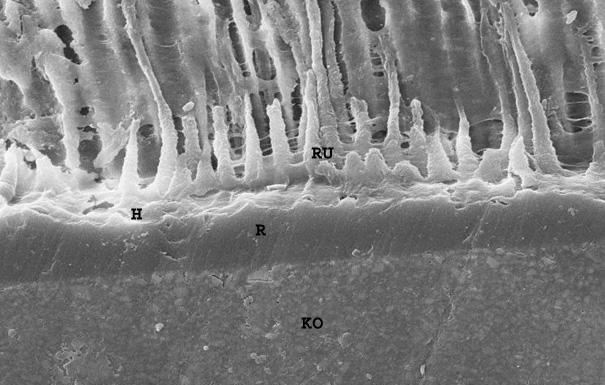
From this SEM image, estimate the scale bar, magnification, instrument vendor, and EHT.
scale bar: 10000 nm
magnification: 1 K X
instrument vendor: Zeiss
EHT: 20 kV